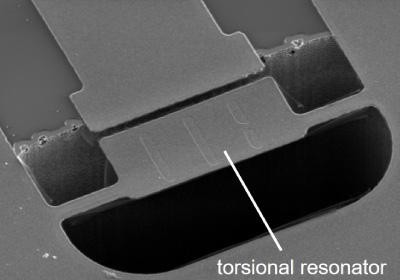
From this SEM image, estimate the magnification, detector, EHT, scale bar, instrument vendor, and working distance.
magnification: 0.6 K X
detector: SE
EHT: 5 kV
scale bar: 50000 nm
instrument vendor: Hitachi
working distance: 34.2 mm